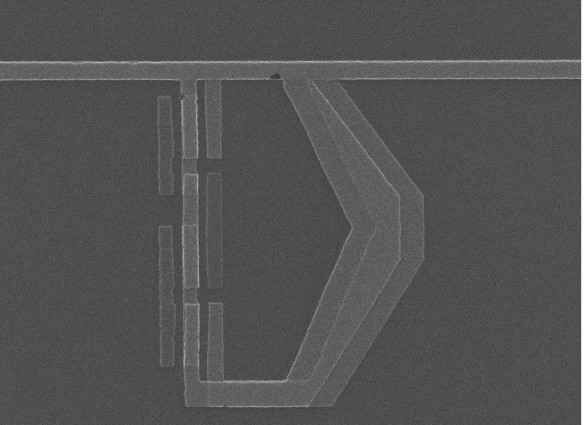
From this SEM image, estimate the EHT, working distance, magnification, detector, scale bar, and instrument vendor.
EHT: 15 kV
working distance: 9.2 mm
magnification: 16 K X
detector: SEI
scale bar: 1000 nm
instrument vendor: JEOL